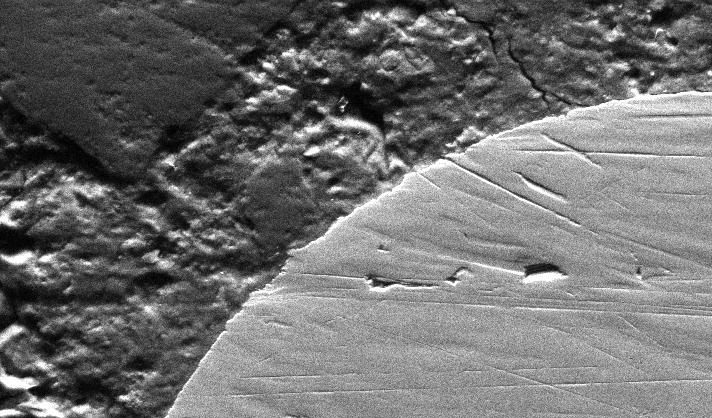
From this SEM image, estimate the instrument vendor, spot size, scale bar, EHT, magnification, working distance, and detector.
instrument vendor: FEI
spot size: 5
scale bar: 100000 nm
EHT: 30 kV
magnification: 0.291 K X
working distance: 7.5 mm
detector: SE